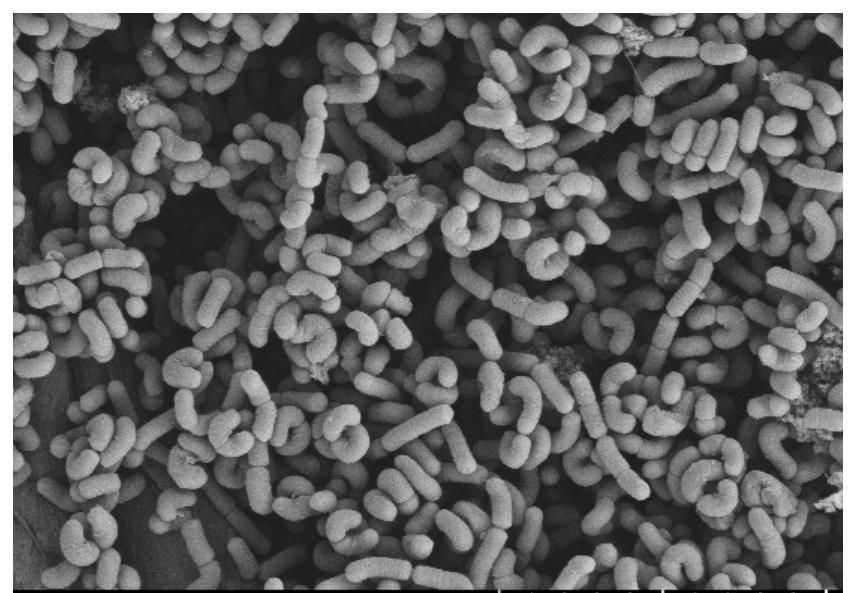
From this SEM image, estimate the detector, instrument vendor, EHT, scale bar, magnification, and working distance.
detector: SE(L)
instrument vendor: Hitachi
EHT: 5 kV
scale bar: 10000 nm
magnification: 5 K X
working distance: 15.2 mm